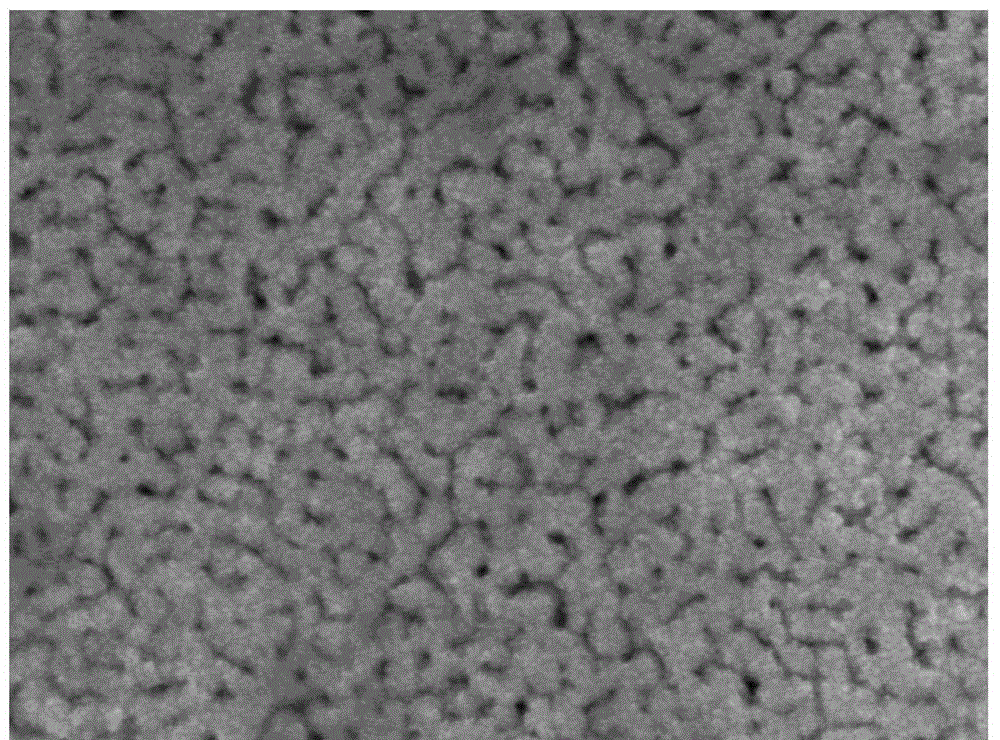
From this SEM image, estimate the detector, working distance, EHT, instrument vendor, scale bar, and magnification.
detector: UED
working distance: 4 mm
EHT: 5 kV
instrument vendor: JEOL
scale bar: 100 nm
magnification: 100 K X